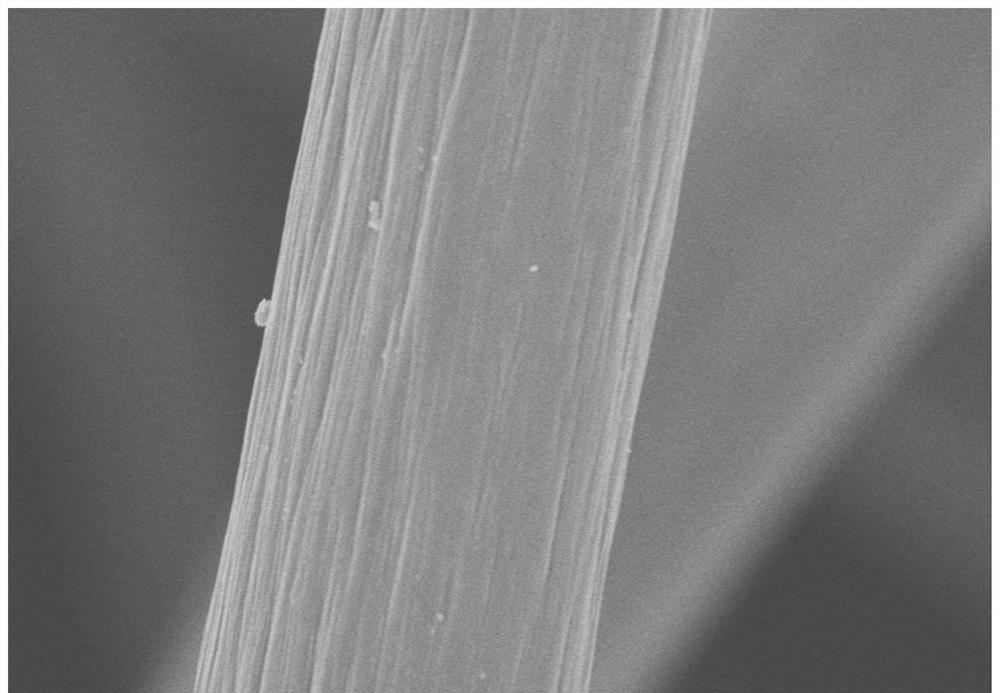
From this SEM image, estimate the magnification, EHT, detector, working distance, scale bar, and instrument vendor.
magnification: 5 K X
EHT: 5 kV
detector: SE(M)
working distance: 9.9 mm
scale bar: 10000 nm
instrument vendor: Hitachi